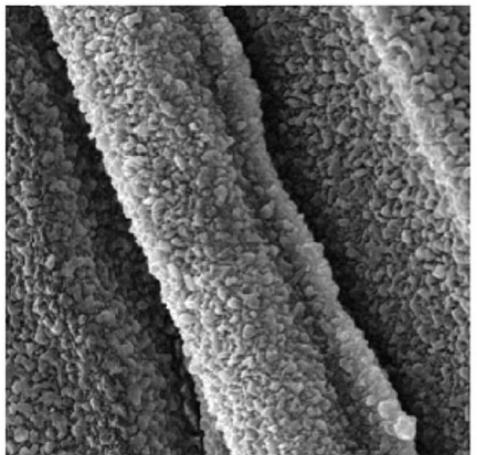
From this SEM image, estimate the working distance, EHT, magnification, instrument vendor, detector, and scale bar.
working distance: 11.61 mm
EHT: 10 kV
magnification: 15.64 K X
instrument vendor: Tescan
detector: SE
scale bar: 2000 nm